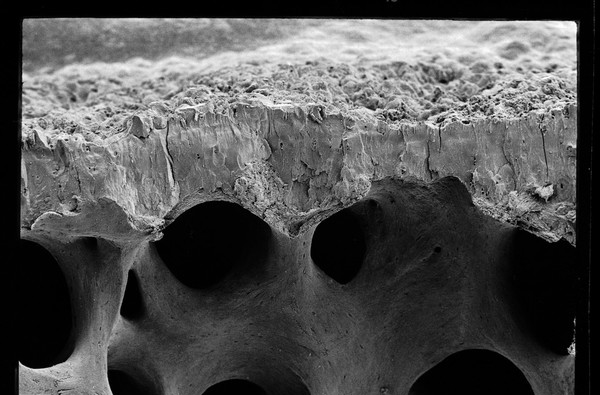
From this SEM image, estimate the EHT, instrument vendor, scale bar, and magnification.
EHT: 10 kV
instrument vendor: JEOL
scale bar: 100000 nm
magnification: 0.054 K X